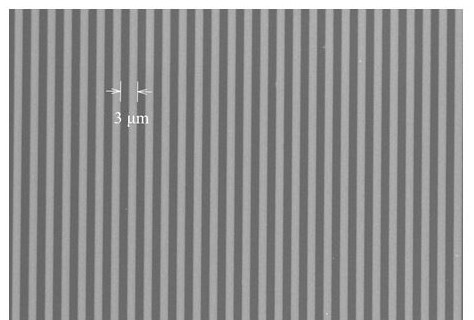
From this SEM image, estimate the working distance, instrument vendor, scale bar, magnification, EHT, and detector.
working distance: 4.1 mm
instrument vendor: Hitachi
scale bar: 30000 nm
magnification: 1.5 K X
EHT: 1 kV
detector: SE+BSE(TU)